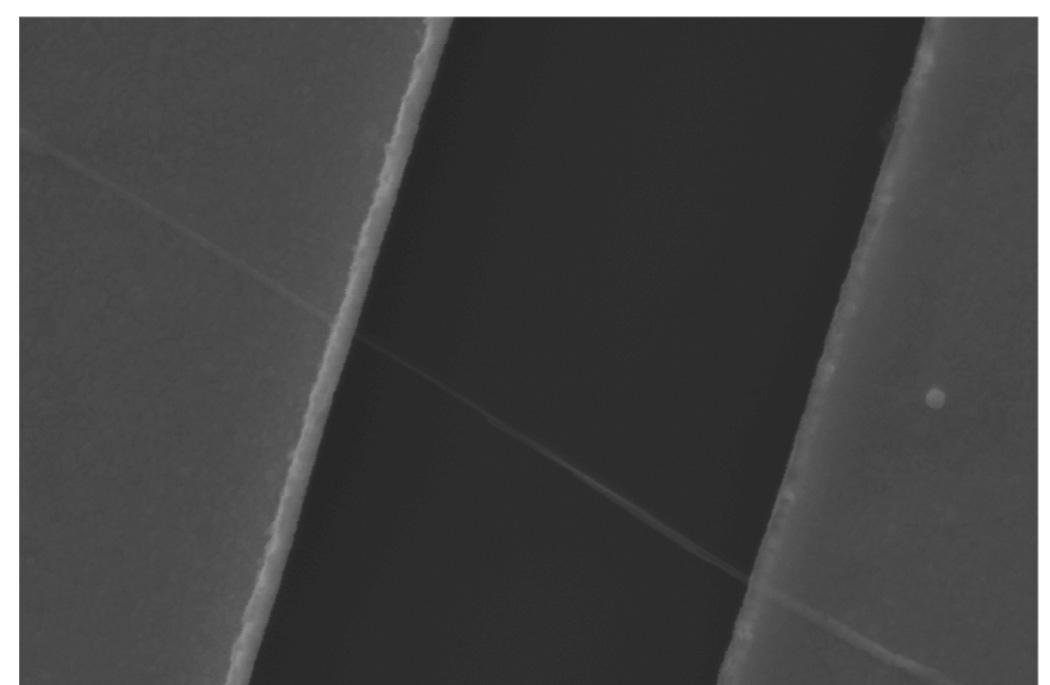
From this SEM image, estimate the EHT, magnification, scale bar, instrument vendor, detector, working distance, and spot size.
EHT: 15 kV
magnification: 24.069 K X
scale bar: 1000 nm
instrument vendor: FEI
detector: SE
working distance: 7.3 mm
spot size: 3